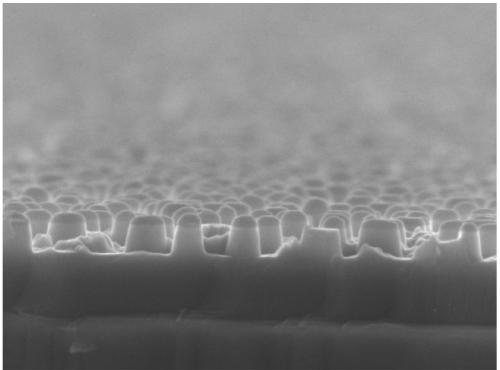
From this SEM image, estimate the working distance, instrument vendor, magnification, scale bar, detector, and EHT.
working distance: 7.9 mm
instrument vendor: JEOL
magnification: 40 K X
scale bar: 100 nm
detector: SEI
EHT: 8 kV